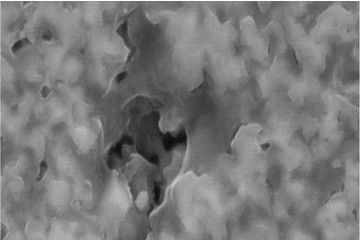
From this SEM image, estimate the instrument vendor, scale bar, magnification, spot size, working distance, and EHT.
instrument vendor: JEOL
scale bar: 600 nm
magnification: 50 K X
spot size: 8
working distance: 5 mm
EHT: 20 kV